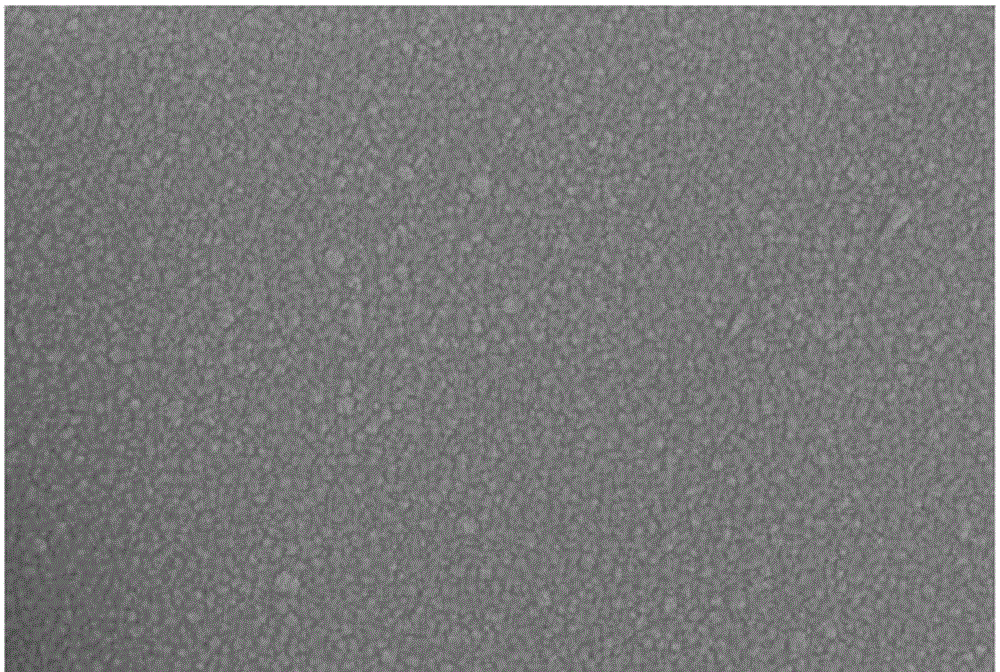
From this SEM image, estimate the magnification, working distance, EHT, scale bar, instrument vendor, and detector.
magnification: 50 K X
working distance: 6.9 mm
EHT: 15 kV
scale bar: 100 nm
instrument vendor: Zeiss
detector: InlensDuo